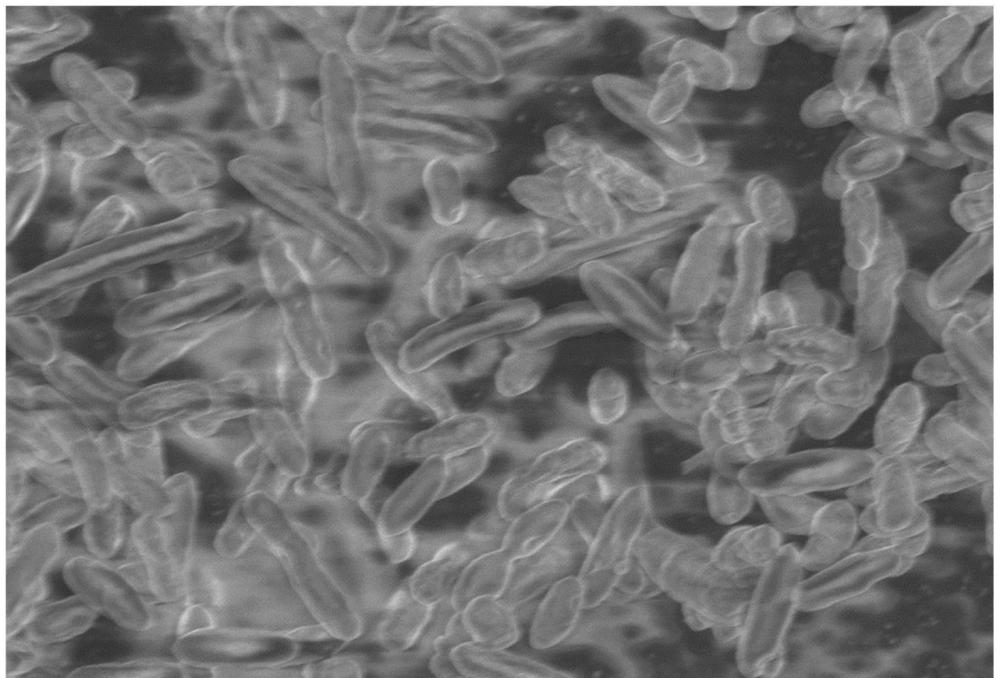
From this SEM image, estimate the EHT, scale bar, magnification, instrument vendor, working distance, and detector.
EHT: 2 kV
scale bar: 2000 nm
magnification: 8.77 K X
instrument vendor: Zeiss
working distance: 6.5 mm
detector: InLens